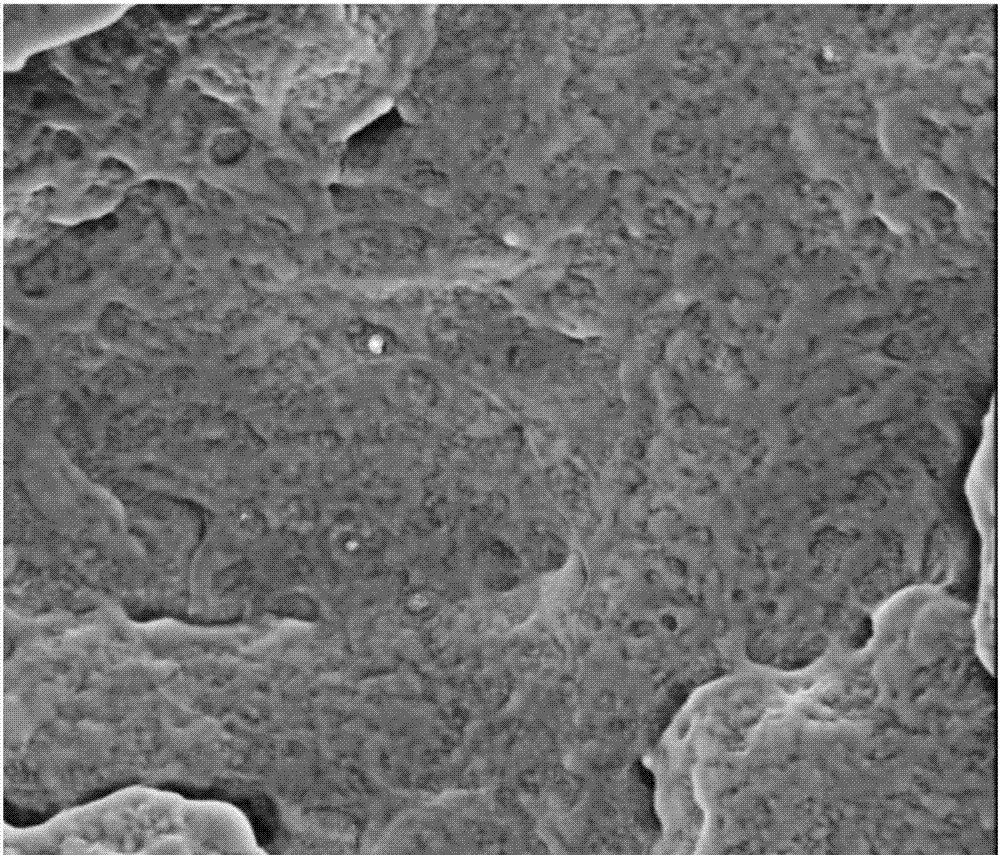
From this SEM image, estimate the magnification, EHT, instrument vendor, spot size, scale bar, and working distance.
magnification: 20 K X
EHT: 3 kV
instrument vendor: FEI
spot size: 3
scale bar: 2000 nm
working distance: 5.3 mm